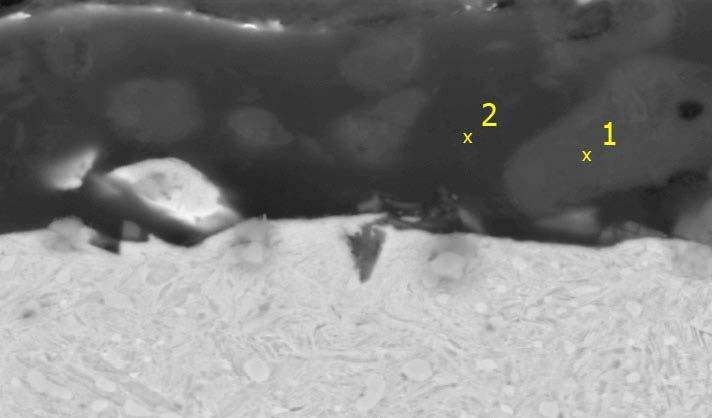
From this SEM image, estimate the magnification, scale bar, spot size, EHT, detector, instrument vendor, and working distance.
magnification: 3 K X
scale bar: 5000 nm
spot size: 4.5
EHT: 20 kV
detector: BSE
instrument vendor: FEI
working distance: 11 mm